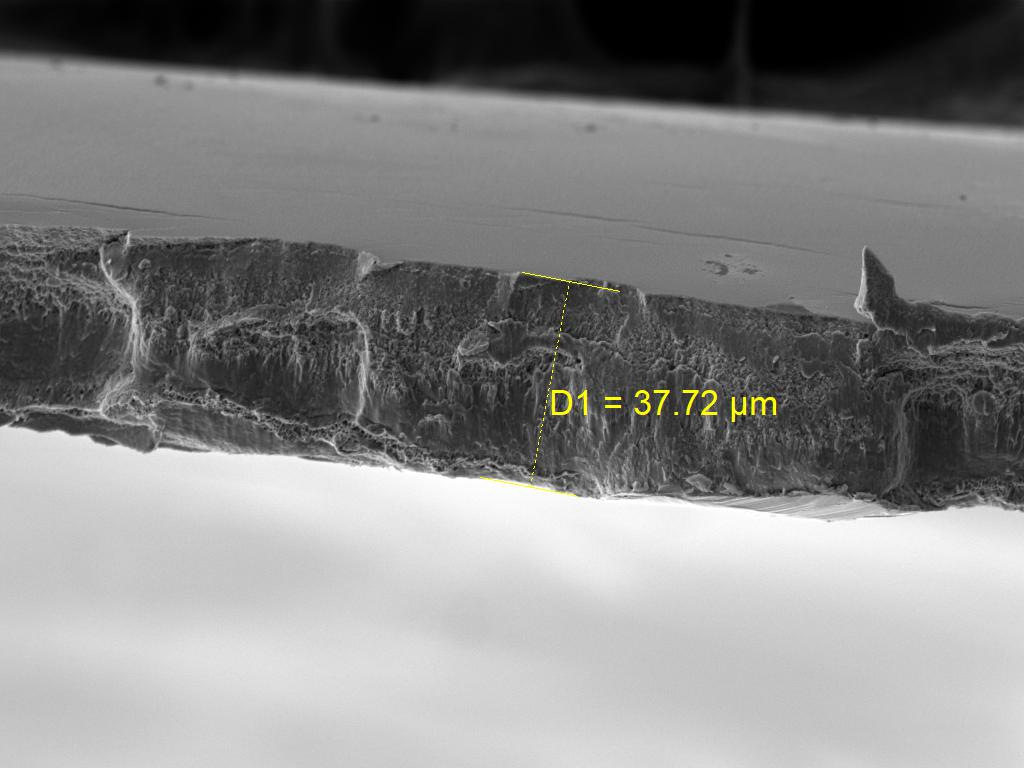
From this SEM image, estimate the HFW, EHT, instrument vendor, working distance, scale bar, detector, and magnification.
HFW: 185 µm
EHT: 15 kV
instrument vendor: Tescan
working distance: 9.7 mm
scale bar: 50000 nm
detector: SE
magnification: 1.5 K X